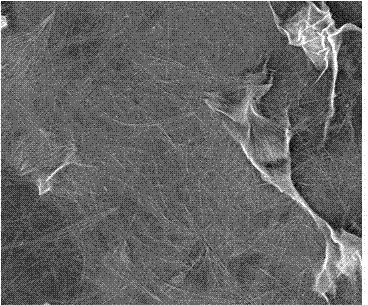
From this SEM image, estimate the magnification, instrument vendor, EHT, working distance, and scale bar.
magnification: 0.8 K X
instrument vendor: FEI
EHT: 10 kV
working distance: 17.1 mm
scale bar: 100000 nm